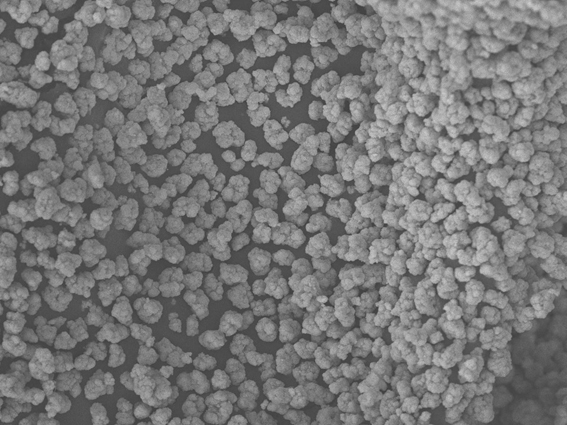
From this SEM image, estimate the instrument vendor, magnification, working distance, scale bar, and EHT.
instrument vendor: JEOL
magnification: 1 K X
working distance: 8 mm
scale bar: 10000 nm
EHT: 20 kV